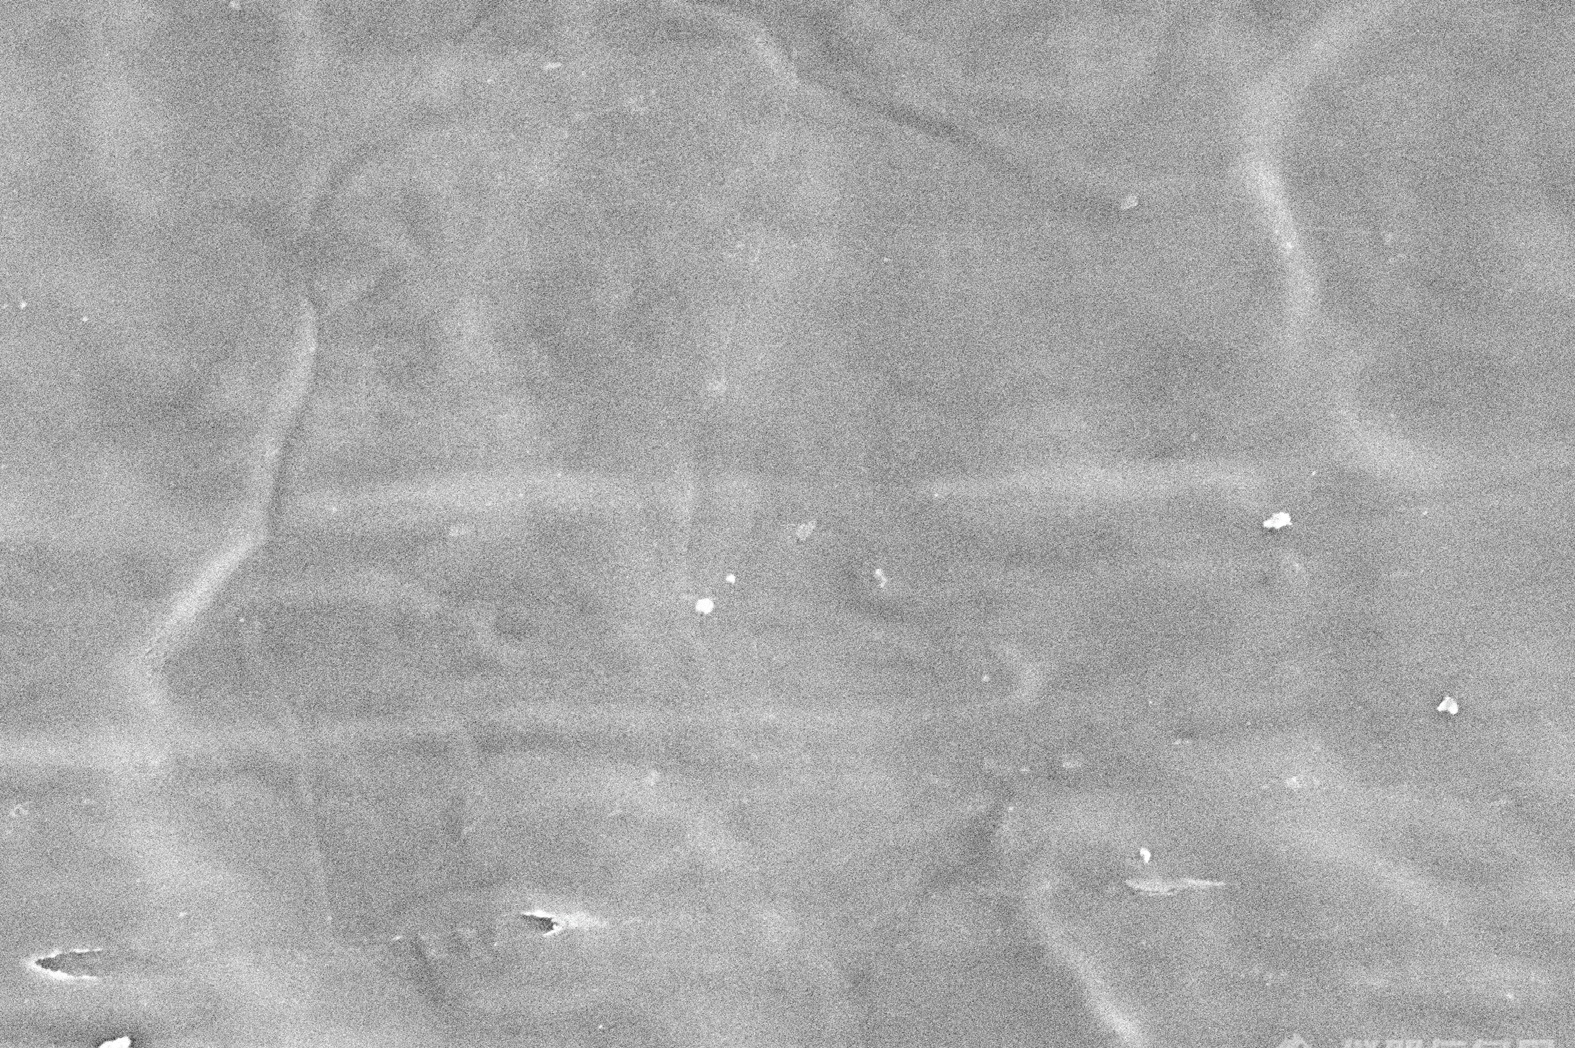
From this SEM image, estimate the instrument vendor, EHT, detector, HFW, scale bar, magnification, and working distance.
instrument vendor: Thermo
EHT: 2 kV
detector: ETD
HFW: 20 µm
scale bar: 5000 nm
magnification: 10.36 K X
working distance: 5 mm